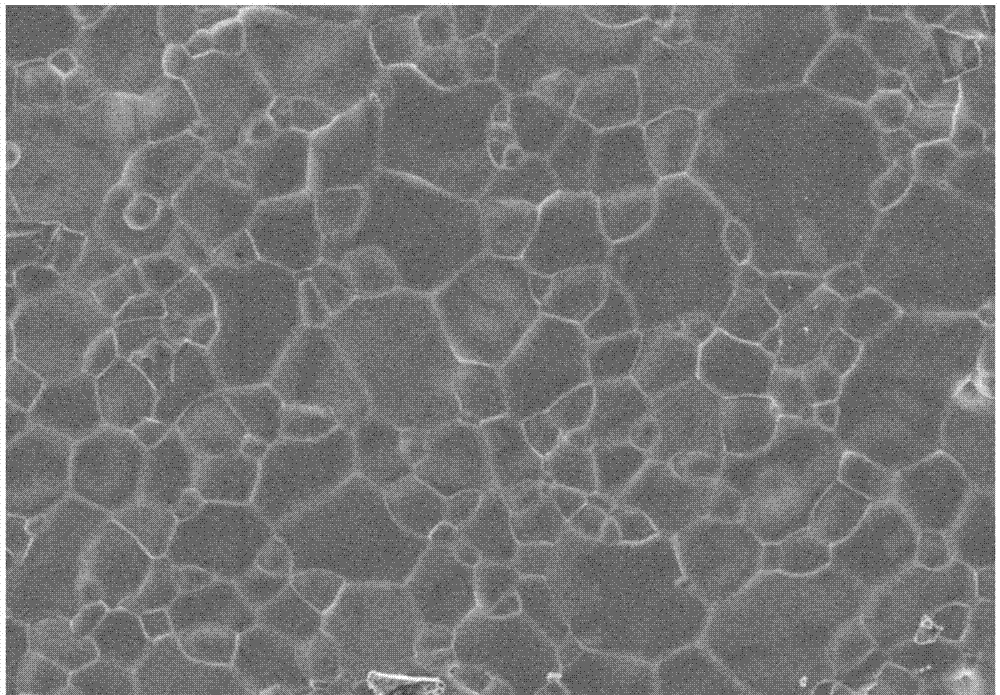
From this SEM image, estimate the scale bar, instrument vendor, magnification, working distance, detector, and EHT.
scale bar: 10000 nm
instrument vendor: Hitachi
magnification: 3 K X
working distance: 10.1 mm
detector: SE(M)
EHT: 3 kV